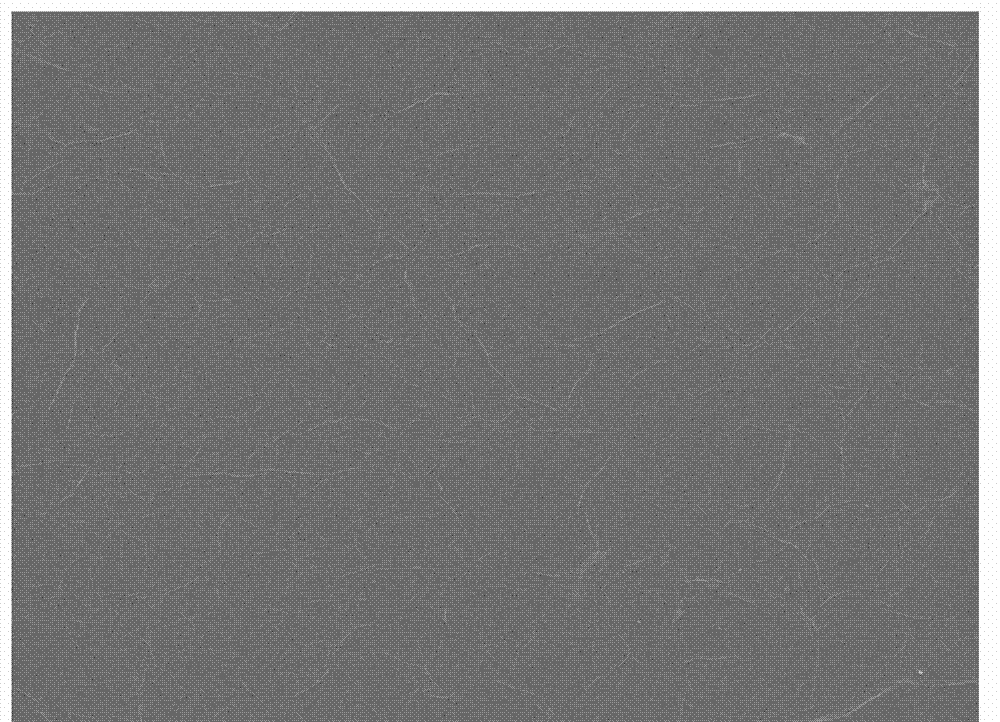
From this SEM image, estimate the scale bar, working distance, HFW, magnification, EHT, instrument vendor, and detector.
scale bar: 5000 nm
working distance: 10 mm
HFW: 27.04 µm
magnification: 10 K X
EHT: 15 kV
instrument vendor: FEI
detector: ETD